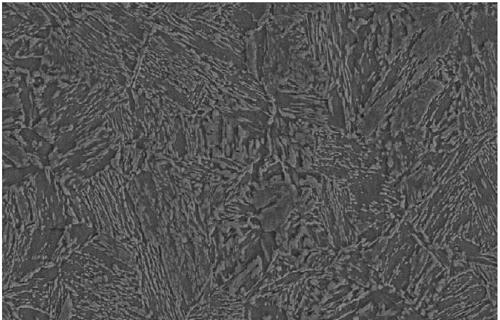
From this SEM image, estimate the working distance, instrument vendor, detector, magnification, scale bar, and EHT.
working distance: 14.3 mm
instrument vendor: Zeiss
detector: SE2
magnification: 2 K X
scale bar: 2000 nm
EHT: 20 kV